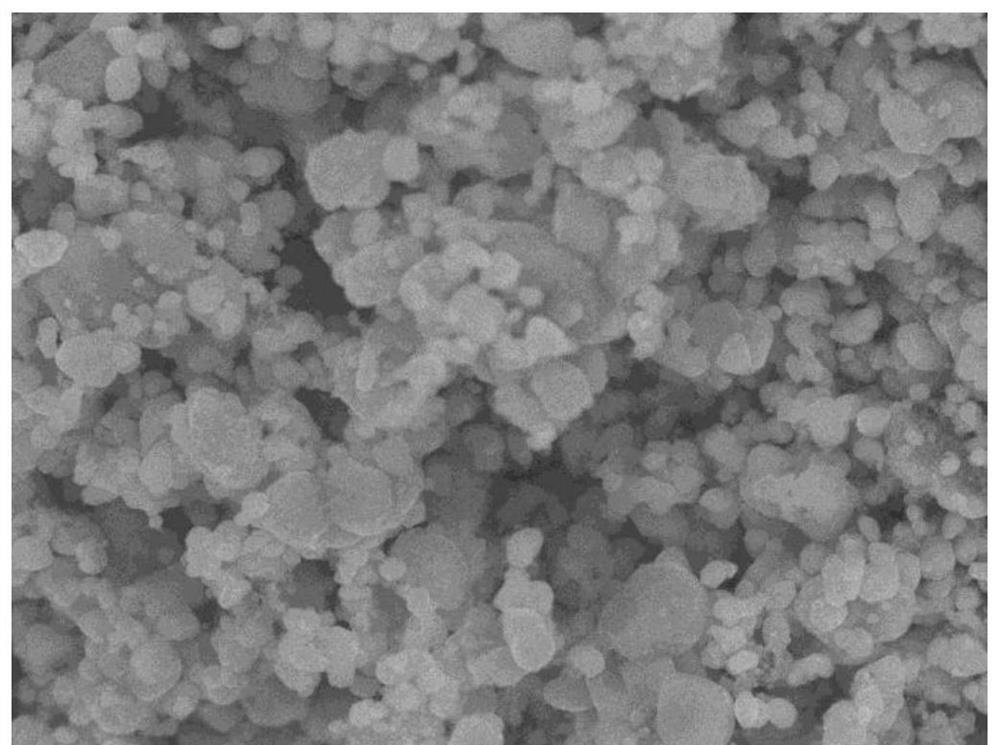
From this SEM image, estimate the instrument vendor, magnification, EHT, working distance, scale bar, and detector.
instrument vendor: JEOL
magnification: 10 K X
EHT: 10 kV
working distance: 7.3 mm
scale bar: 1000 nm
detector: SEI